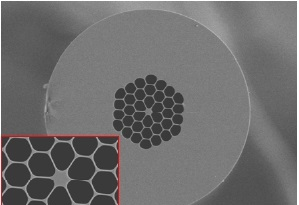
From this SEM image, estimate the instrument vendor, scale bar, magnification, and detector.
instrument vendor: Hitachi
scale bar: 50000 nm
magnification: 0.7 K X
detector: SE(L)